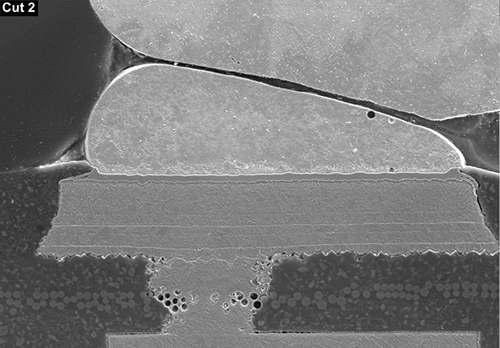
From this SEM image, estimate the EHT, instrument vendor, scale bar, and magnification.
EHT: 20 kV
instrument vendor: JEOL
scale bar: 28600 nm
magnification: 0.35 K X